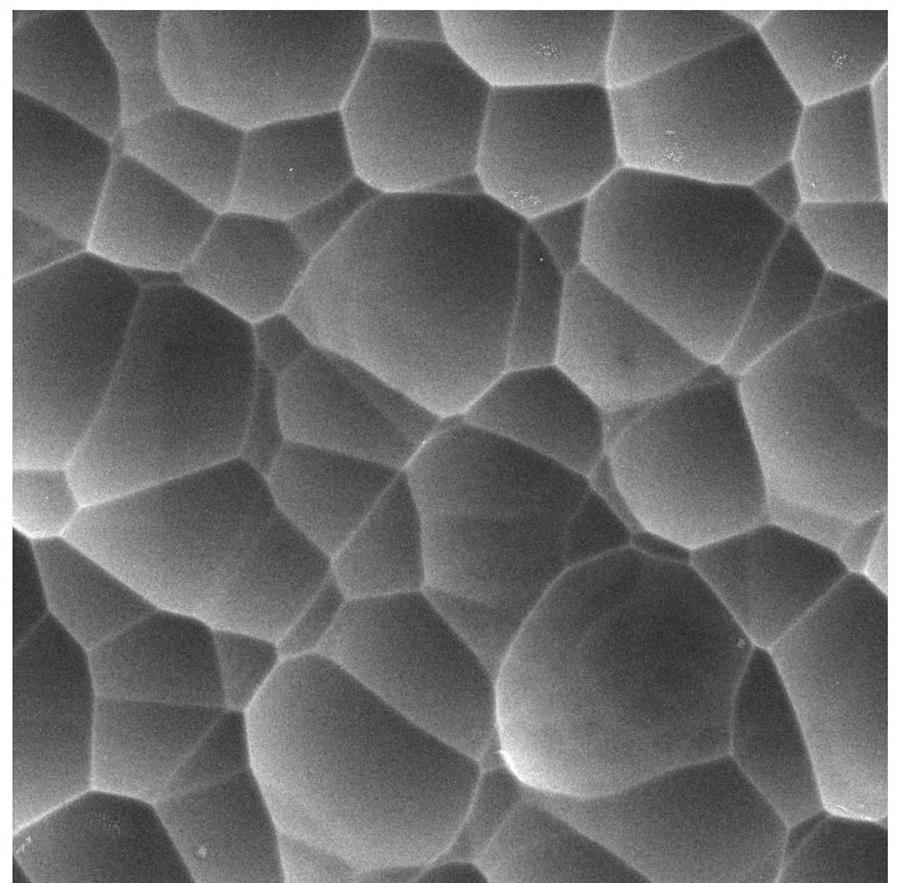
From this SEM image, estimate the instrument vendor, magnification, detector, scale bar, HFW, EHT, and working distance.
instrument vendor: Tescan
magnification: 10 K X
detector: SE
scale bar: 5000 nm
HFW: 20.8 µm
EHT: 5 kV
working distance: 10.14 mm